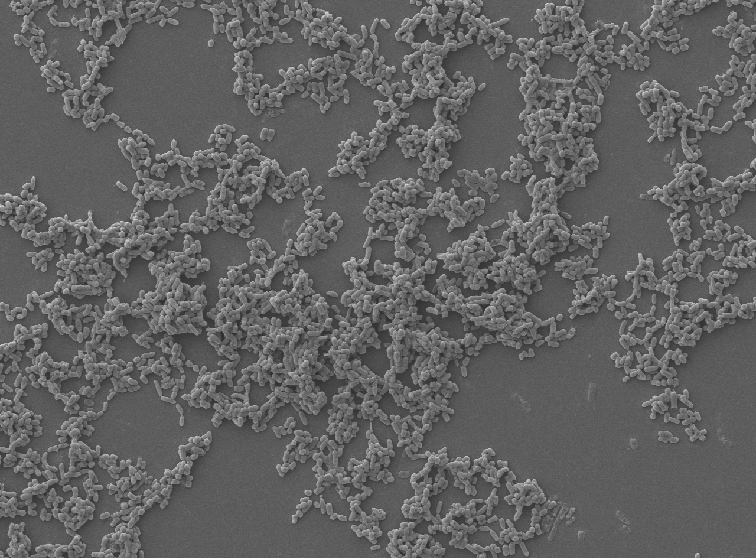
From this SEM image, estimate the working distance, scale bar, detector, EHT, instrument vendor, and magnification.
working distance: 10.8 mm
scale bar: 10000 nm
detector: SED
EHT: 5 kV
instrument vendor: JEOL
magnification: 1.5 K X